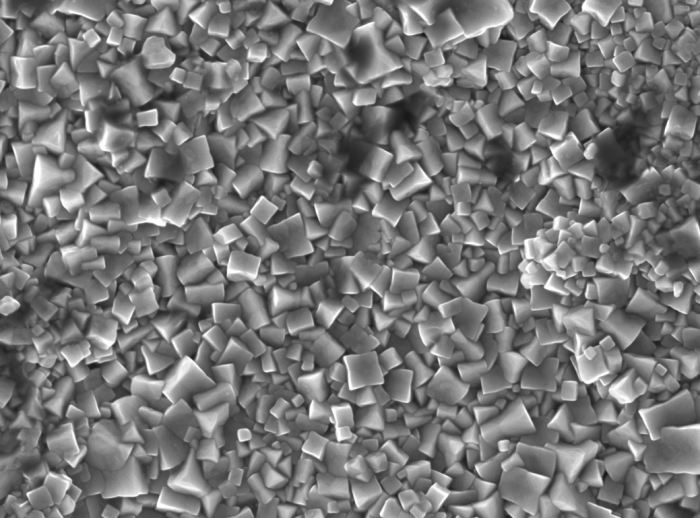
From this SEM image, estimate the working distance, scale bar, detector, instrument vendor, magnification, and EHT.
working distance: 8 mm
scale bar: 1000 nm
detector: SEI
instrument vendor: JEOL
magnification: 10 K X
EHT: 10 kV